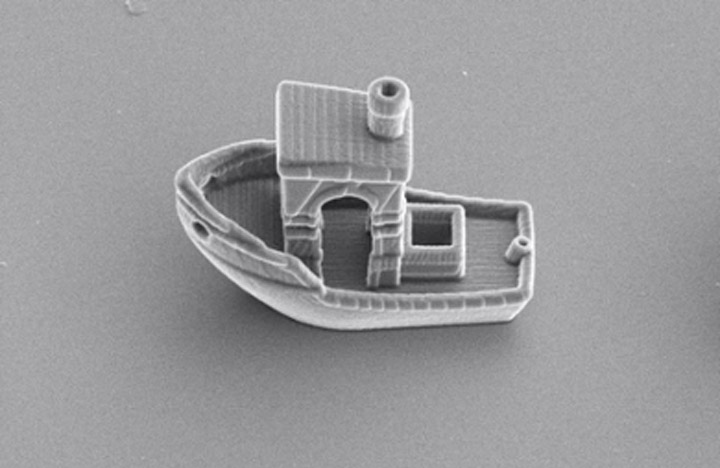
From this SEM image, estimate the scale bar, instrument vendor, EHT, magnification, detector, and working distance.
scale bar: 20000 nm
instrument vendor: Thermo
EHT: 5 kV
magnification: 3.5 K X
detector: ETD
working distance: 12.9 mm